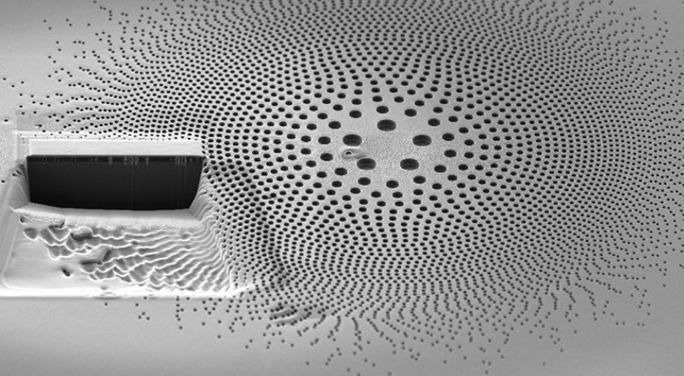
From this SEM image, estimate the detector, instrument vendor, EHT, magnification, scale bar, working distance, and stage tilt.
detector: SE2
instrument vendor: Zeiss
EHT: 2 kV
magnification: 1.99 K X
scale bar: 2000 nm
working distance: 4.9 mm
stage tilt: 54°